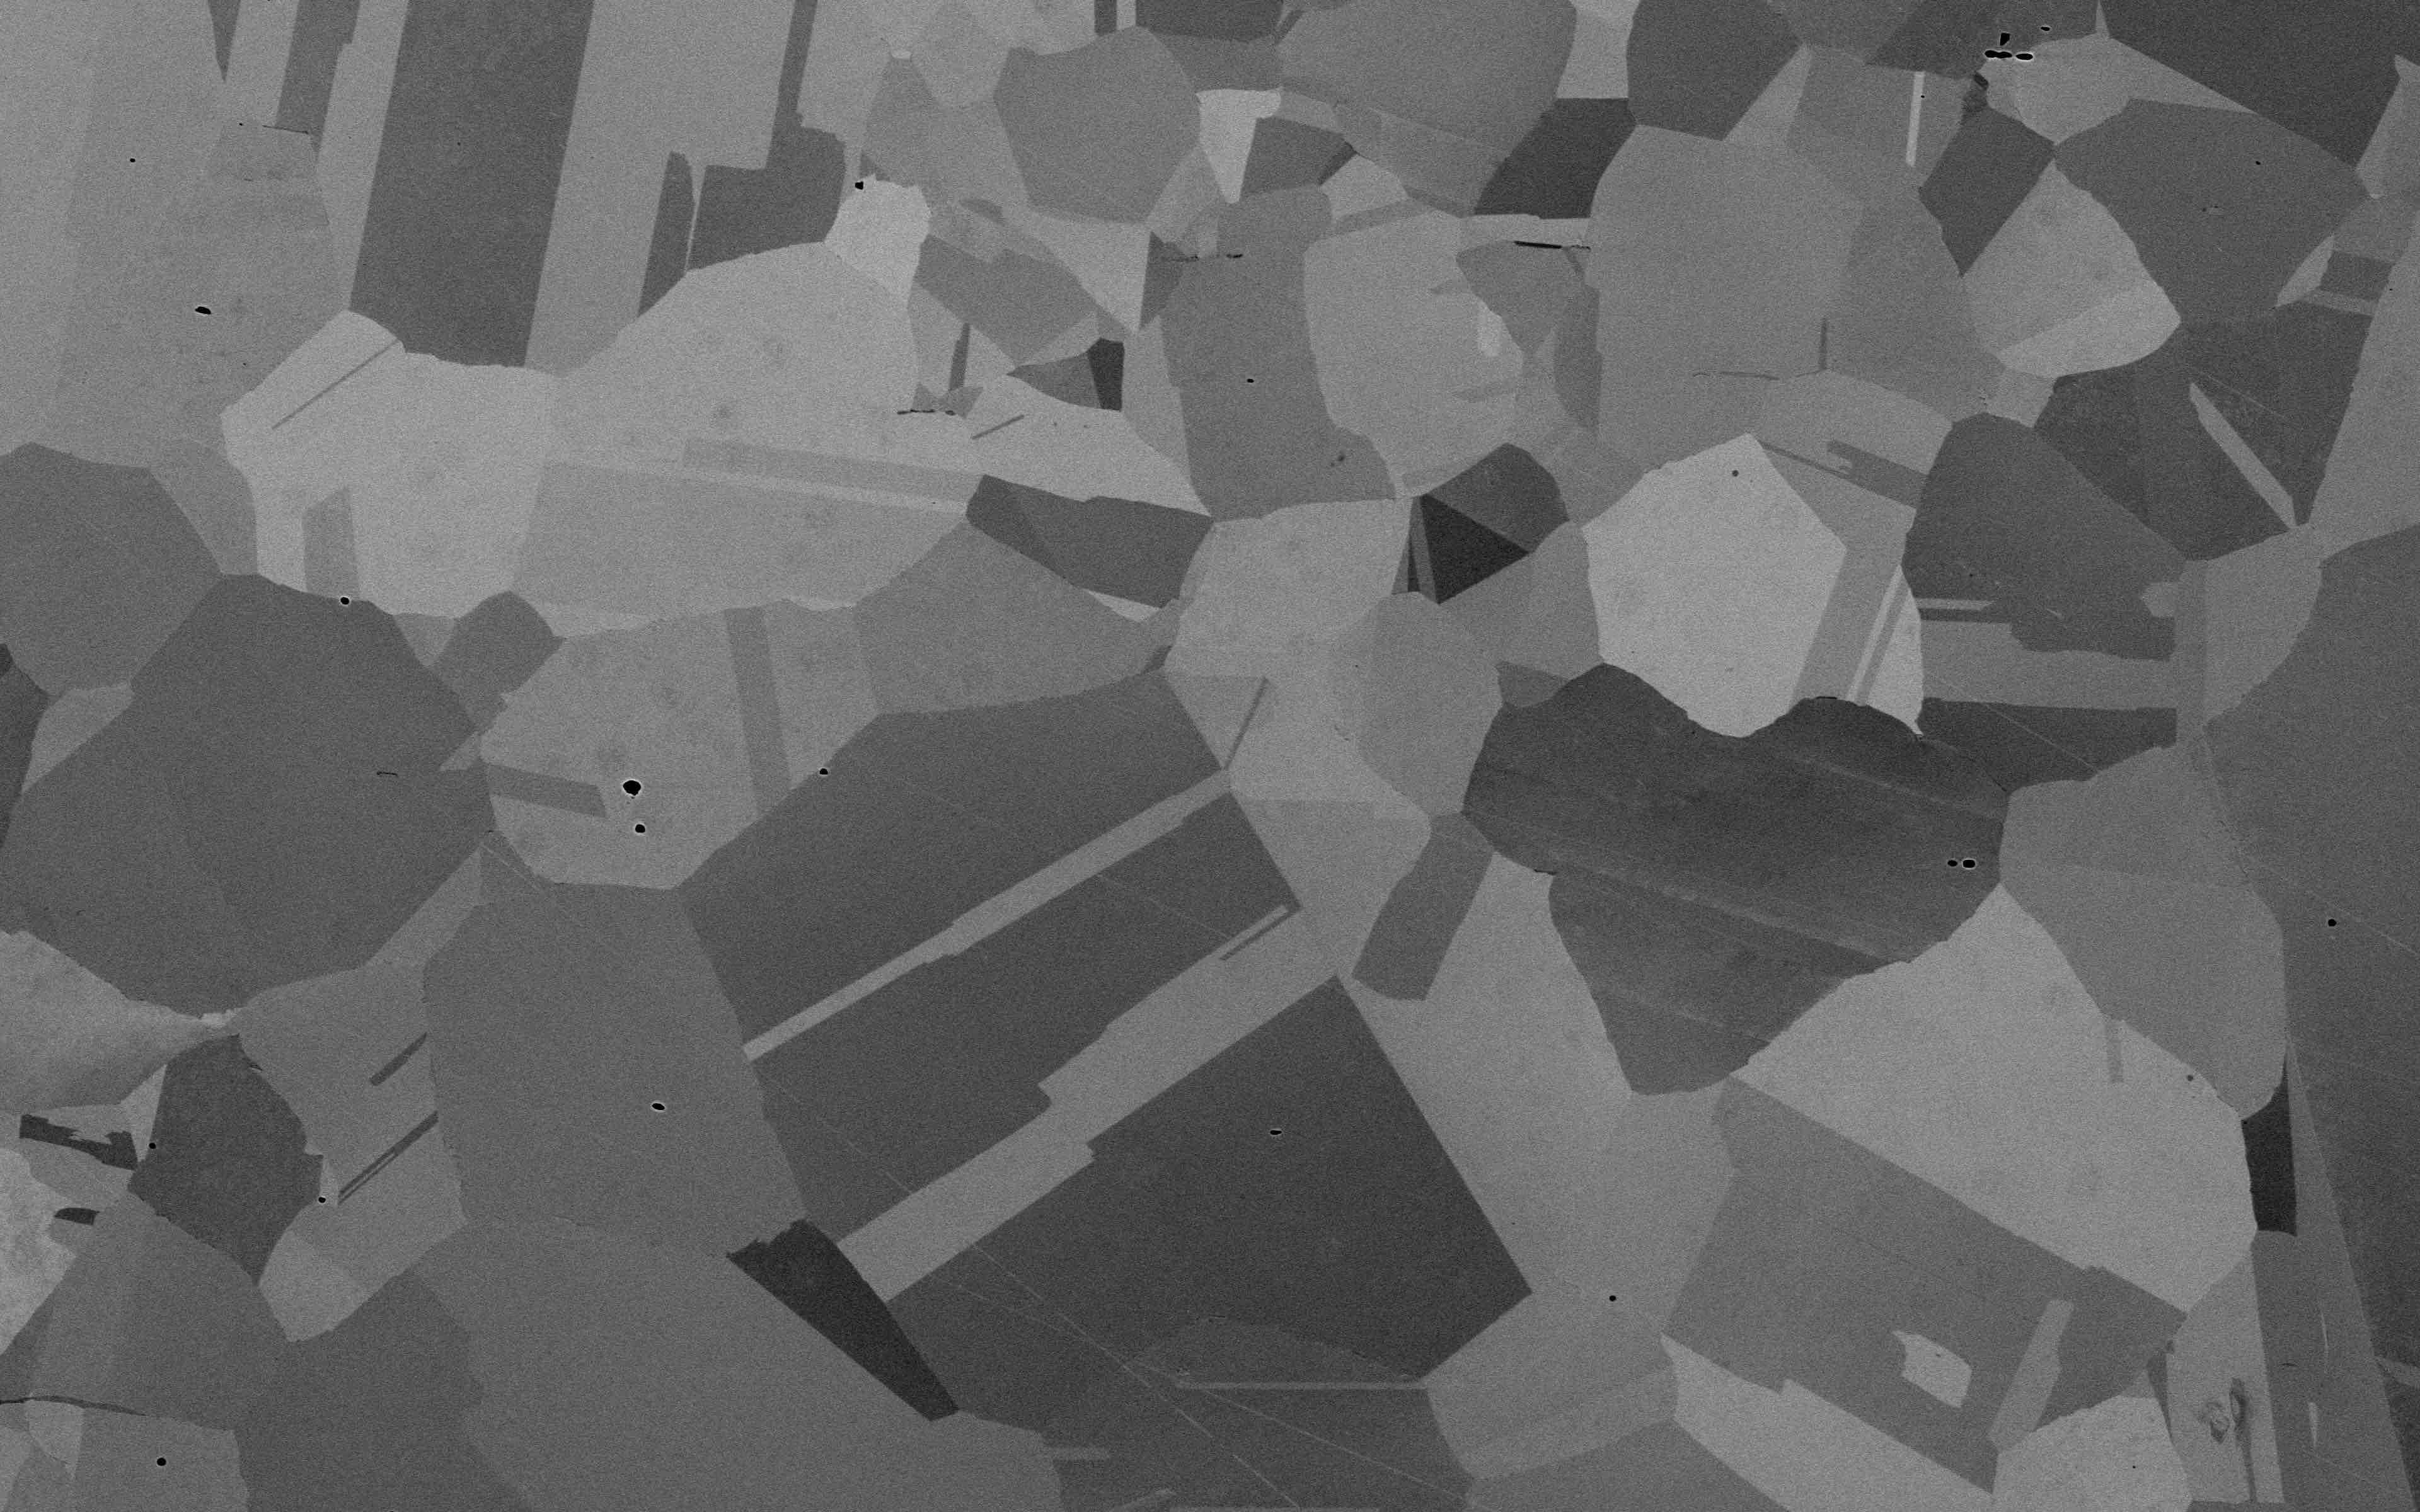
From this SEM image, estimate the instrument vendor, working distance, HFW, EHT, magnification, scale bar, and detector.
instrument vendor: Thermo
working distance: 8.863 mm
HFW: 508 µm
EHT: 20 kV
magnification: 0.25 K X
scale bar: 150000 nm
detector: BSD Full